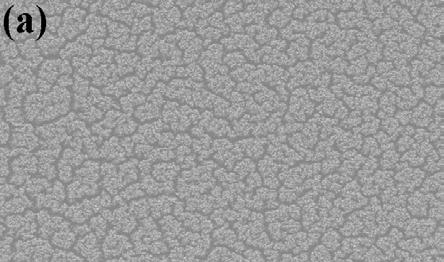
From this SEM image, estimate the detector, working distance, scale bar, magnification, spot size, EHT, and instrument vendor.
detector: SE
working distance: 25.3 mm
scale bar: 200000 nm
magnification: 0.1 K X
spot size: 4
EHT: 20 kV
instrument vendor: FEI